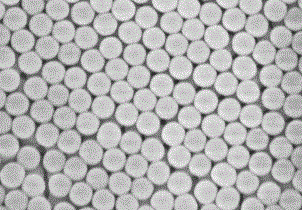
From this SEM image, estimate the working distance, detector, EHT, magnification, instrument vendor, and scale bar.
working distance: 8.7 mm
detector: SE(M)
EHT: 5 kV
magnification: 40 K X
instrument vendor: Hitachi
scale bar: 1000 nm